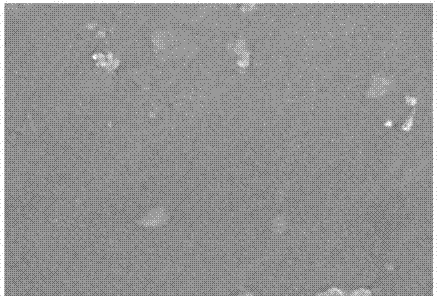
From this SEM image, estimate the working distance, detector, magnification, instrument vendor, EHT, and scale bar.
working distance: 2.1 mm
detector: InLens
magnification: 50 K X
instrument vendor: Zeiss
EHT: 3 kV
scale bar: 100 nm